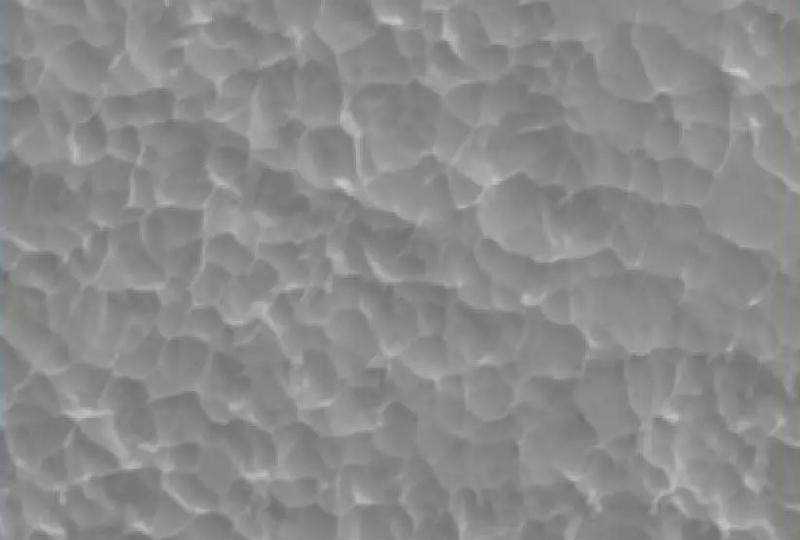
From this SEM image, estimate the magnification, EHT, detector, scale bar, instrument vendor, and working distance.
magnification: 10 K X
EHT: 5 kV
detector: SE2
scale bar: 1000 nm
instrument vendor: Zeiss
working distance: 8.7 mm